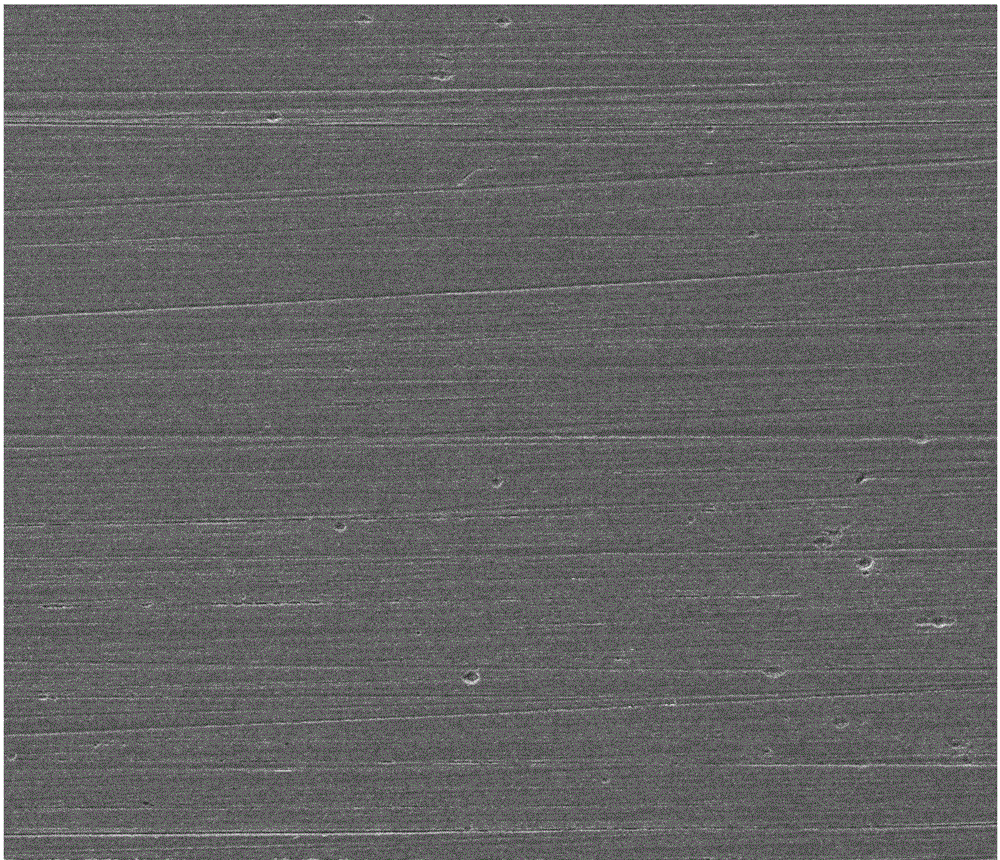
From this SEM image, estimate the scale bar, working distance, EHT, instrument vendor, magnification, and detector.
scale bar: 200000 nm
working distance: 8.8 mm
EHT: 30 kV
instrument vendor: FEI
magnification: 0.5 K X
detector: SE